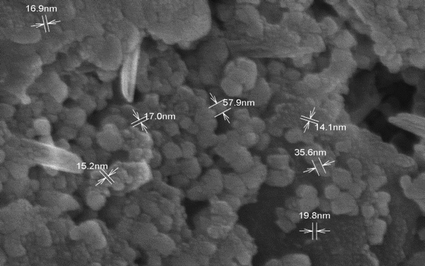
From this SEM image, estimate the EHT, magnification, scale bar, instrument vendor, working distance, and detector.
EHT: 15 kV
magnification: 60 K X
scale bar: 500 nm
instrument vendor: Hitachi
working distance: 13.7 mm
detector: SE(M)